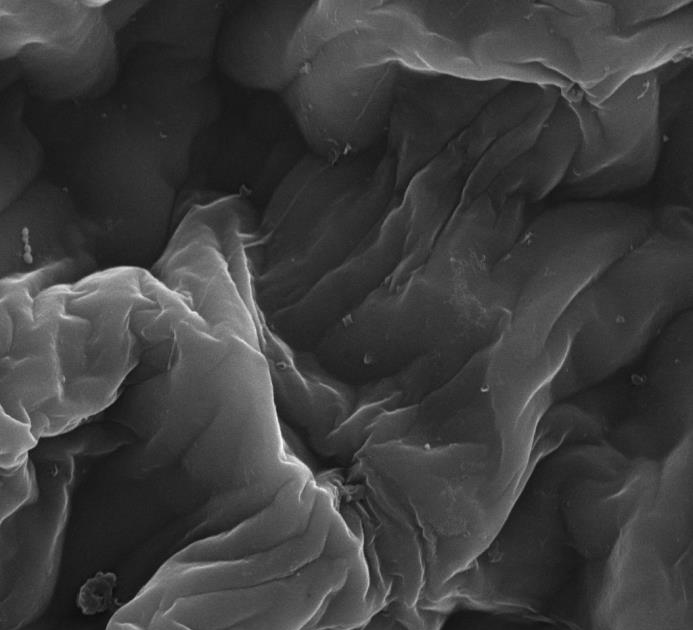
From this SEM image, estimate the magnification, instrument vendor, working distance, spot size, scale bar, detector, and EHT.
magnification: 10 K X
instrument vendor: FEI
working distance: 16.2 mm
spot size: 3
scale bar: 10000 nm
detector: ETD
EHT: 20 kV